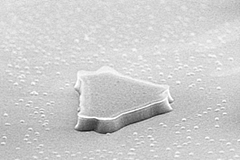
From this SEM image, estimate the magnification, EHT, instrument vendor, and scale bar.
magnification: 10 K X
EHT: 10 kV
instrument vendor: Hitachi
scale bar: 5000 nm